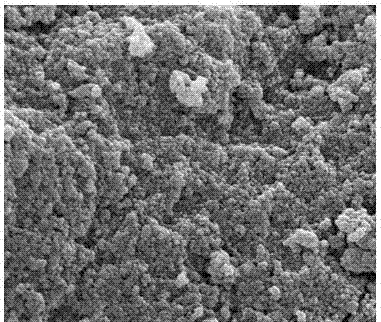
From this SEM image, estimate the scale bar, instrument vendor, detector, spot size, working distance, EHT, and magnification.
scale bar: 3000 nm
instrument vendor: FEI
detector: ETD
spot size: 2.5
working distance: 9.4 mm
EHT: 20 kV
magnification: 30.844 K X